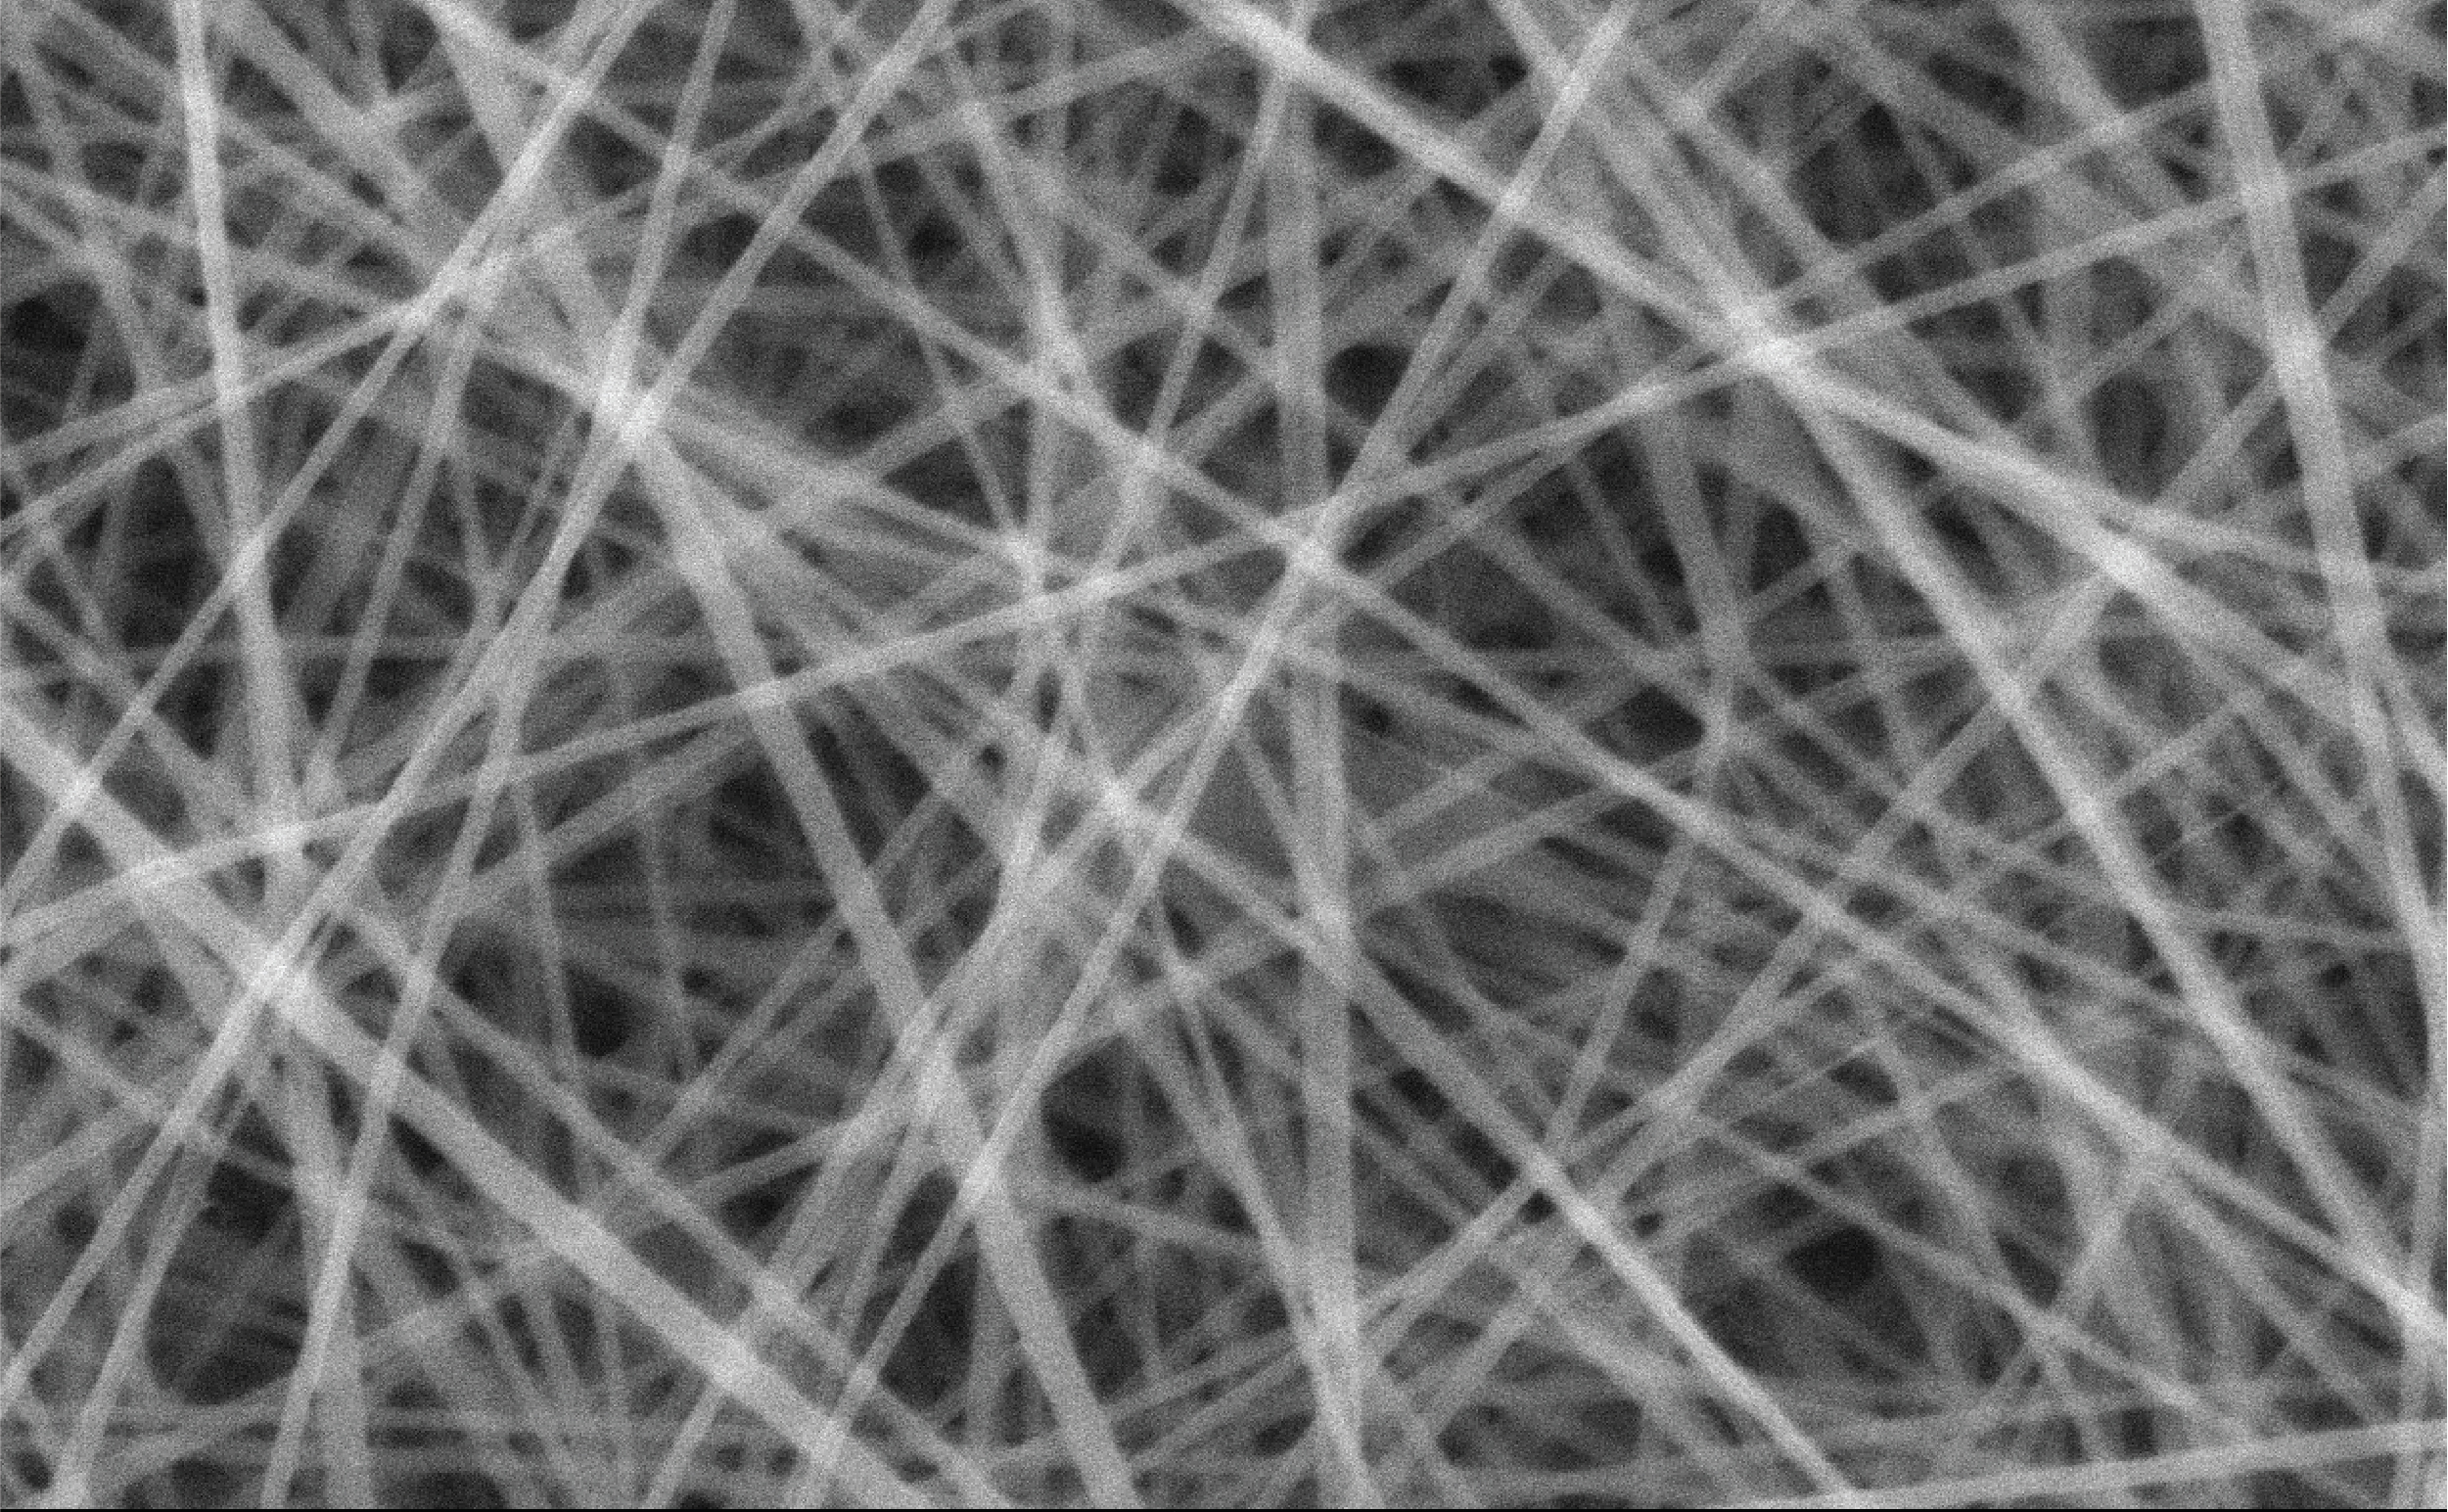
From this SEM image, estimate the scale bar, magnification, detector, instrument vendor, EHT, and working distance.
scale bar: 1000 nm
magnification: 10 K X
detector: SED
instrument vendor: JEOL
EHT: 15 kV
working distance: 10.9 mm